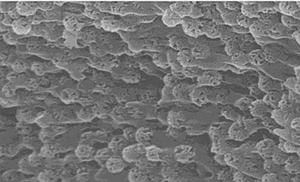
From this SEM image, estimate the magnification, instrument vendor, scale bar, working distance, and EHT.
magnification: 50 K X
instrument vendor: Hitachi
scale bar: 1000 nm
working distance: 9 mm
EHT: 10 kV